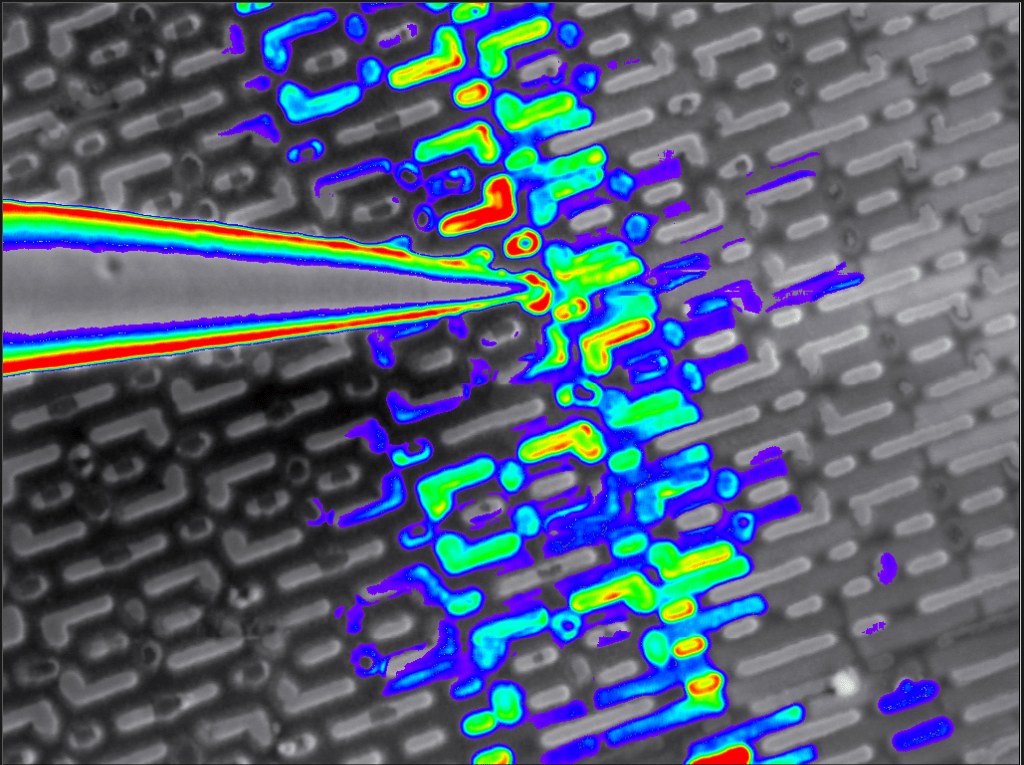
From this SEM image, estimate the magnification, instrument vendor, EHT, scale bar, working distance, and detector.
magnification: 70 K X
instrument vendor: Zeiss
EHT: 0.2 kV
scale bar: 200 nm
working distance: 2.5 mm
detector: MIX: EBIC 0,00+ SE 1,00